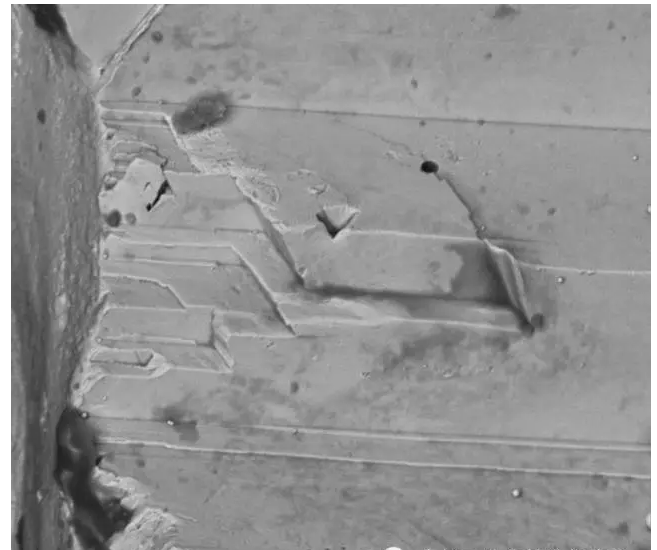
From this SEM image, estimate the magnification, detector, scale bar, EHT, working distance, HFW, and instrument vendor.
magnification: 1.2 K X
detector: SSD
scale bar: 50000 nm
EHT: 25 kV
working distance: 17.3 mm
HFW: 230 µm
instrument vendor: FEI